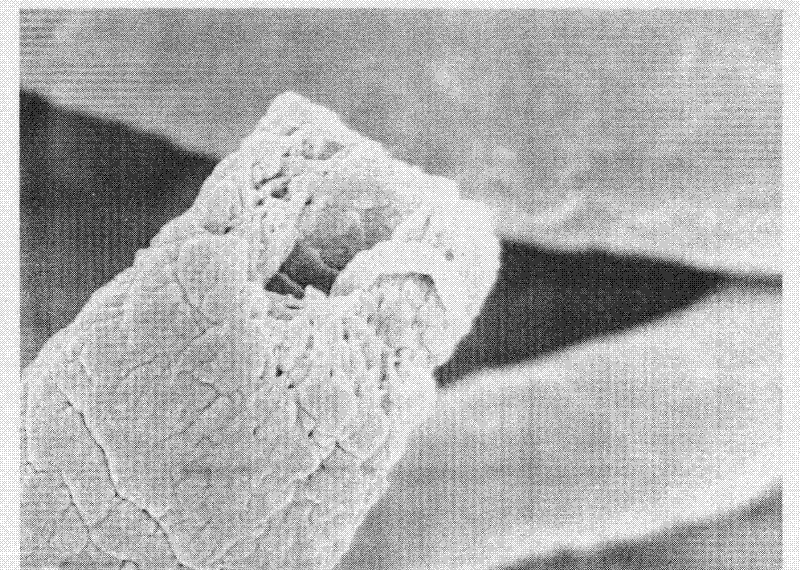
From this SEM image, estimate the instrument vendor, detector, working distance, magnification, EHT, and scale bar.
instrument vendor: JEOL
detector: SEI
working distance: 5.5 mm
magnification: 55 K X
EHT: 3 kV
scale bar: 100 nm